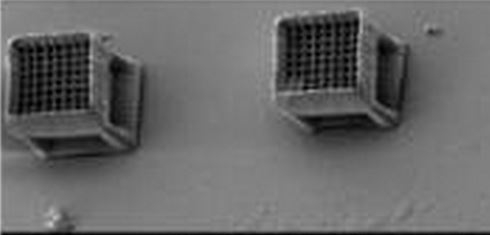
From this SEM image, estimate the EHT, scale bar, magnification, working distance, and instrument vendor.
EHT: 8 kV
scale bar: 100000 nm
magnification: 0.16 K X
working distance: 15 mm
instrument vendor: JEOL